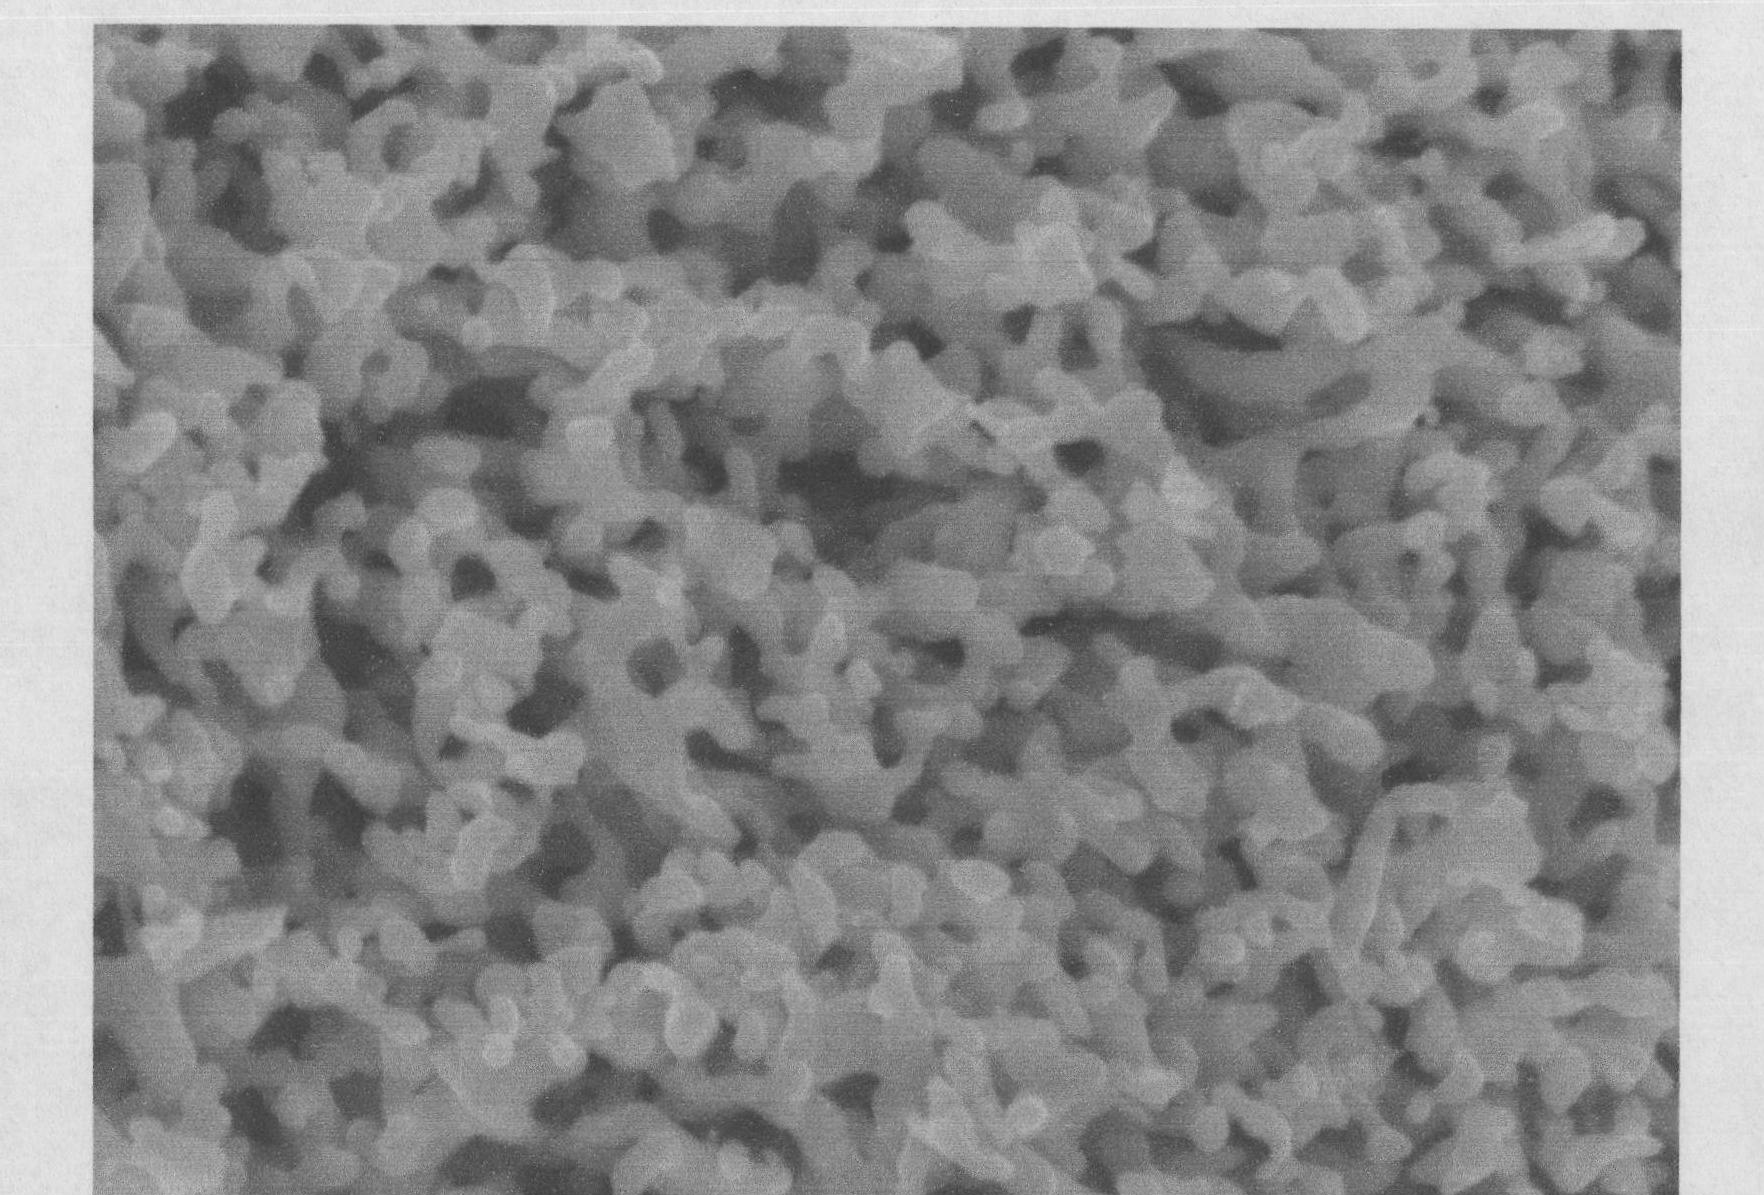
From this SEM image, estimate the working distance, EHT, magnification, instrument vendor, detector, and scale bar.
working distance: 7.9 mm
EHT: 10 kV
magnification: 40 K X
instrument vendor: JEOL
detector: SEI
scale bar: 100 nm